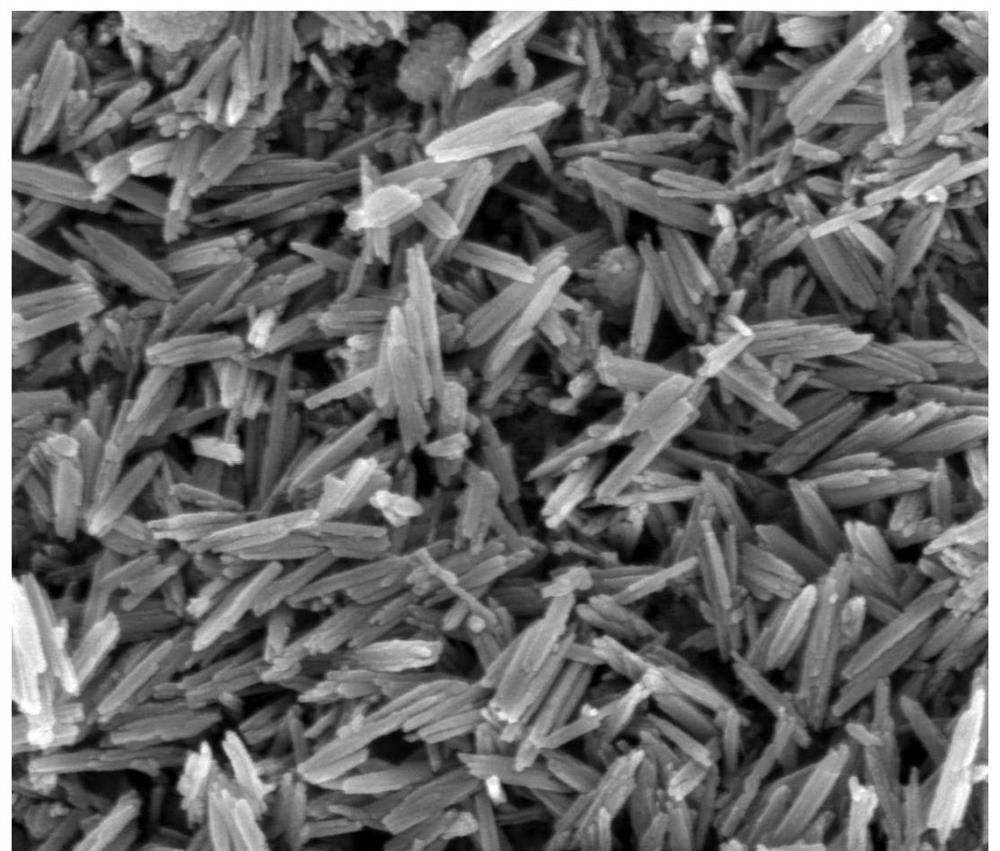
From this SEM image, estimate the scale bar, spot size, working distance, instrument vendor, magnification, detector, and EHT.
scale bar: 500 nm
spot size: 3.5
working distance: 5 mm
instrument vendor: FEI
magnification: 100 K X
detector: TLD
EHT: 10 kV